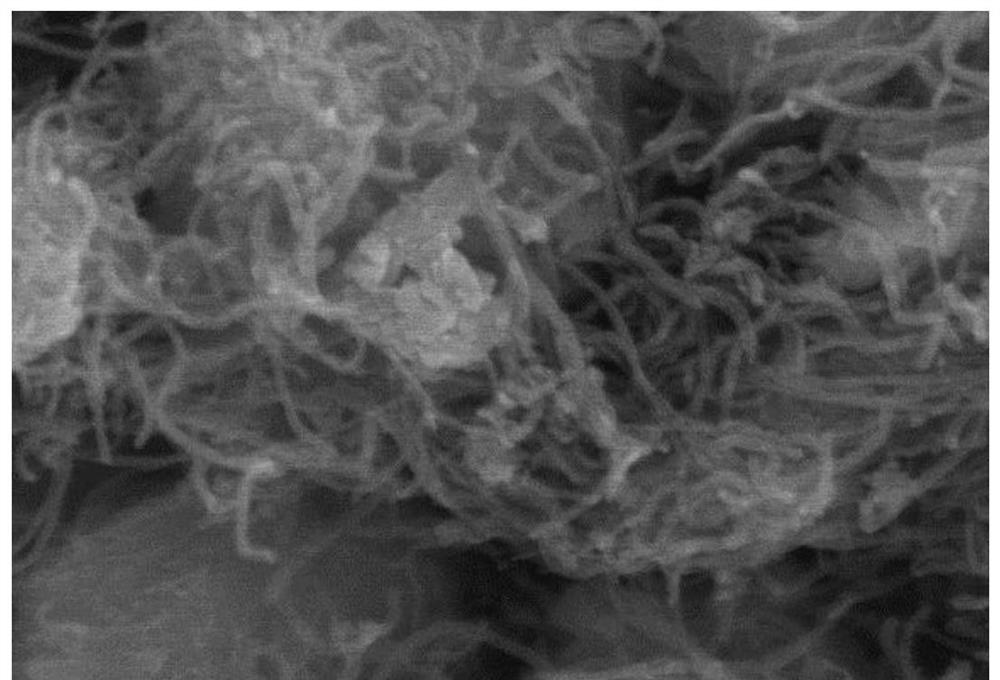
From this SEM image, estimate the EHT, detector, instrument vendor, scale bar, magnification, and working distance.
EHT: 8 kV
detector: SE(UL)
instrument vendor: Hitachi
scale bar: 500 nm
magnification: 100 K X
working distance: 9.7 mm